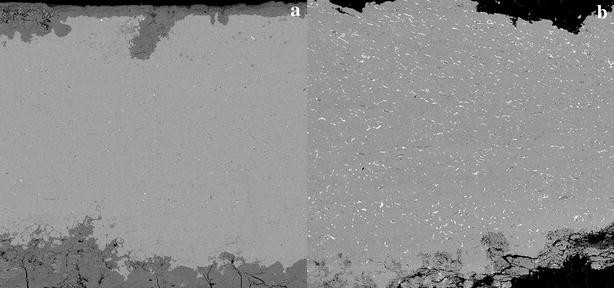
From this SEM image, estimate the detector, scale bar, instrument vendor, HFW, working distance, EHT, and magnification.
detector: BSE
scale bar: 200000 nm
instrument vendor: Tescan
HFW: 722 µm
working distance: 16.71 mm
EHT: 15 kV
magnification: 0.2 K X